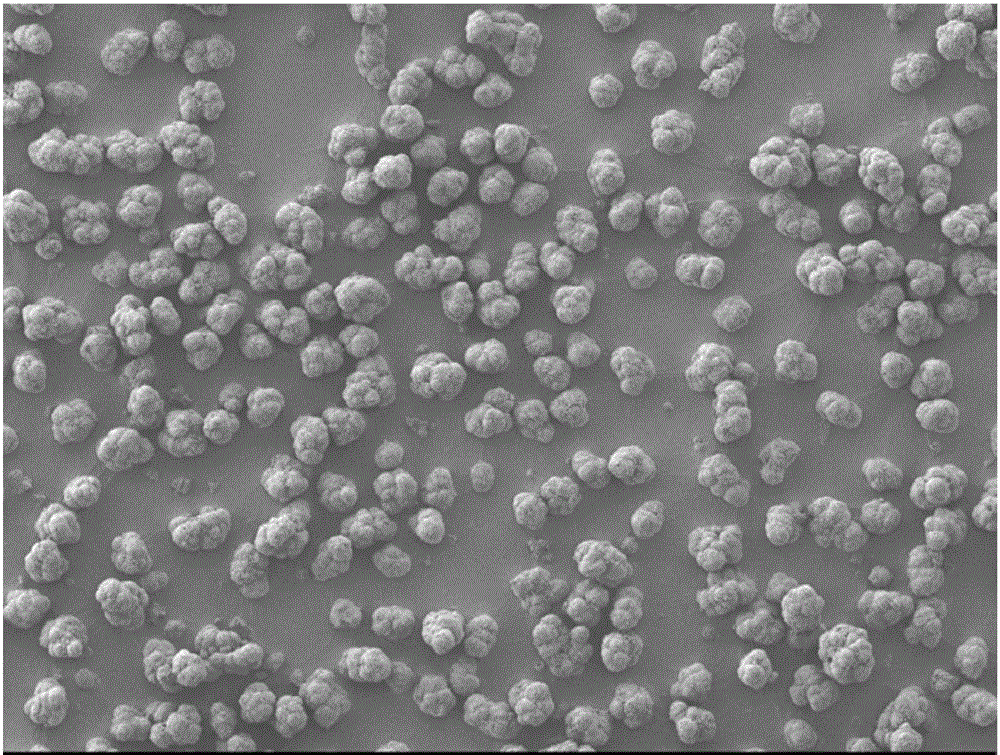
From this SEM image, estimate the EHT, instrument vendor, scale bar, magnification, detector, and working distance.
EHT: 5 kV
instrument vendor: JEOL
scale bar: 100000 nm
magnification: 0.1 K X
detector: LEI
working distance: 8 mm